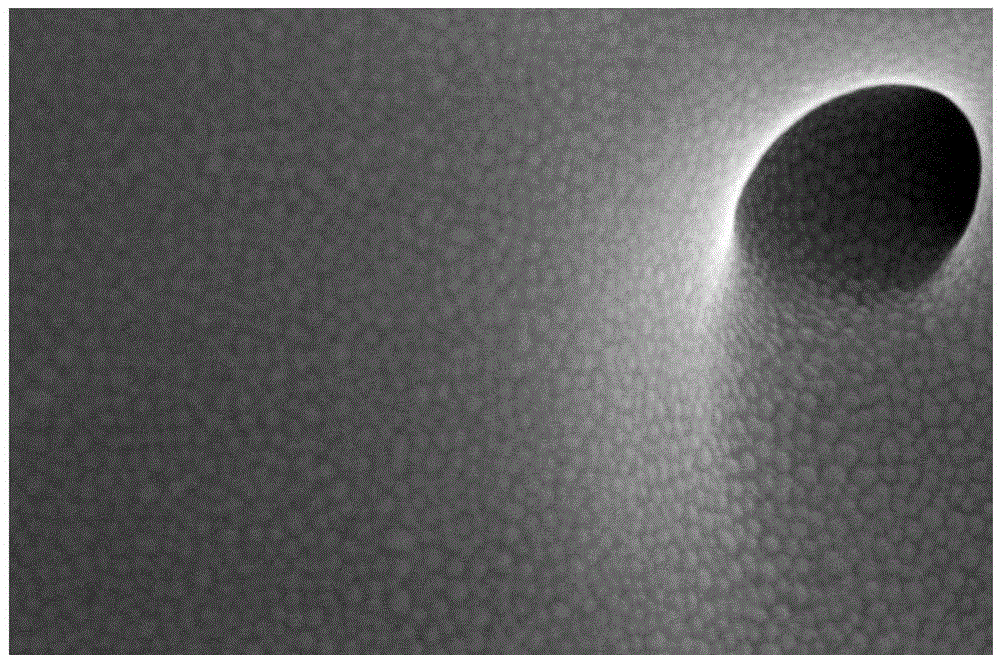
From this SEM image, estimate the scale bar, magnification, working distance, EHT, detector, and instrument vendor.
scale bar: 300 nm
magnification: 500 K X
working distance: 4.7 mm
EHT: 5 kV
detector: TLD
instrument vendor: FEI